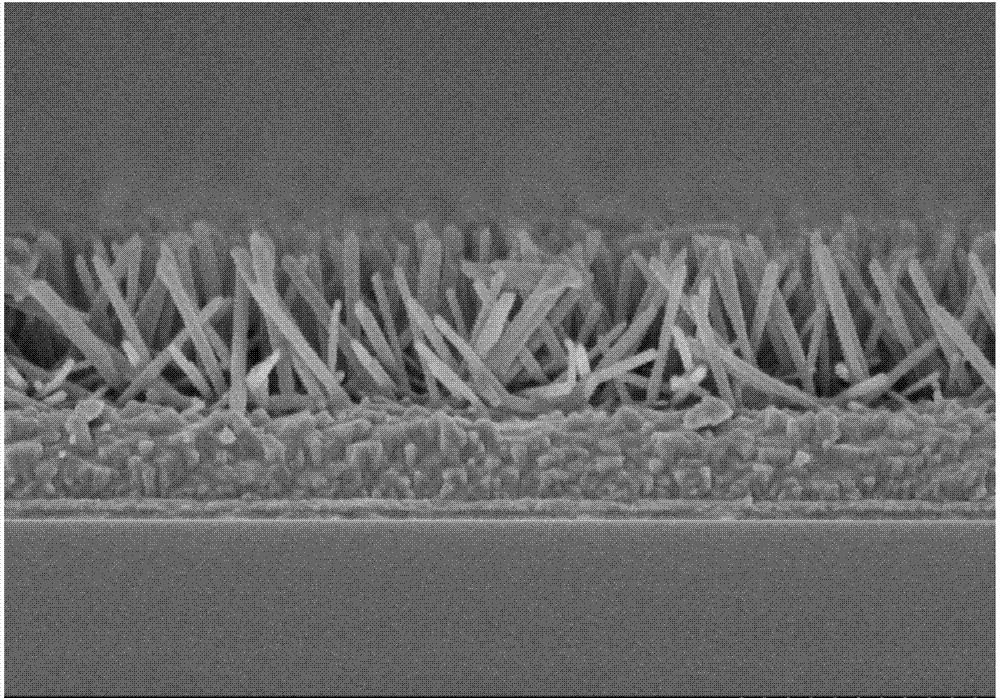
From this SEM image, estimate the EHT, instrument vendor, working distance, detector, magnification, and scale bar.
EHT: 3 kV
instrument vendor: Hitachi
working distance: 8.9 mm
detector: SE(M)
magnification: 30 K X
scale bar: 1000 nm